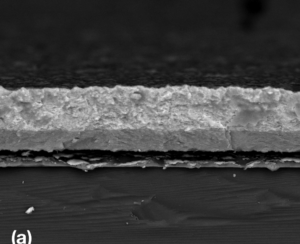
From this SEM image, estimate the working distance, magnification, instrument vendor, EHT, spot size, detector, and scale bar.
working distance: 10.2 mm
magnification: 4 K X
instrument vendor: FEI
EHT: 20 kV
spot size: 3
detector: SSD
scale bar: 20000 nm